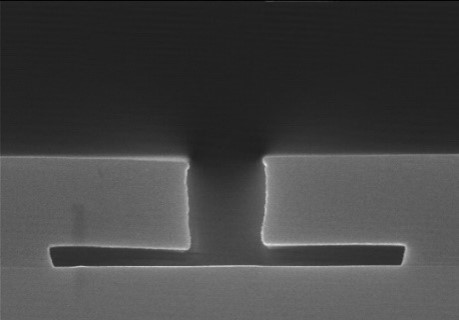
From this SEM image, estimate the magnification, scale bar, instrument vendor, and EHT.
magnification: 30 K X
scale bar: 1000 nm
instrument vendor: Hitachi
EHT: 15 kV